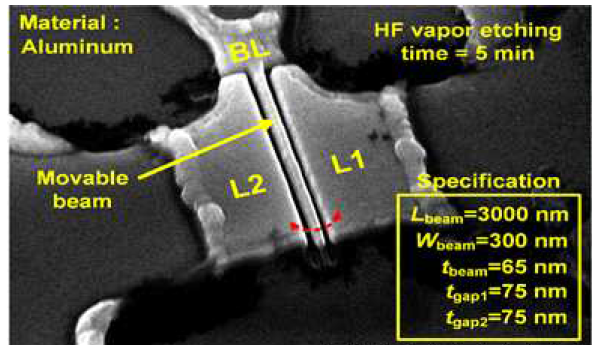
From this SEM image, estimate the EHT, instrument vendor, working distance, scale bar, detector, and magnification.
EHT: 15 kV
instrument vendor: Hitachi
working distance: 10.6 mm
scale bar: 3000 nm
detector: SE(M)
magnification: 18 K X